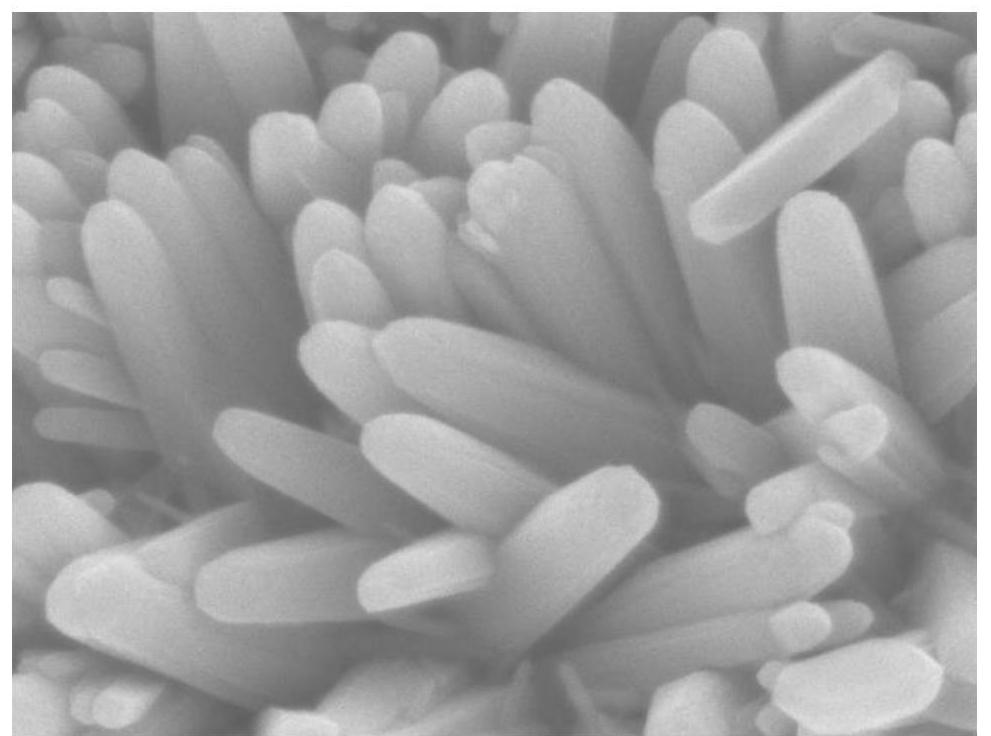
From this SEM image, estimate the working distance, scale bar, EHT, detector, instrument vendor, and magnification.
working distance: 10.5 mm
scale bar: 100 nm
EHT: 10 kV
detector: SEI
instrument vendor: JEOL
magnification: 30 K X